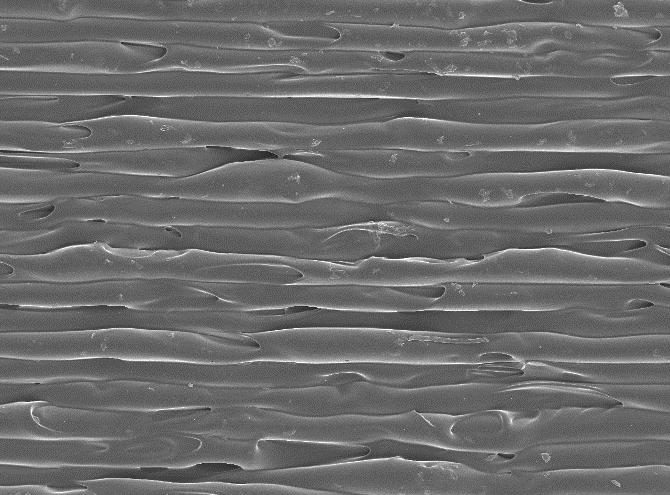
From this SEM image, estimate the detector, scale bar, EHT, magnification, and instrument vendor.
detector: SEI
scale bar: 100000 nm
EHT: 15 kV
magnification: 0.05 K X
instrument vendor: JEOL